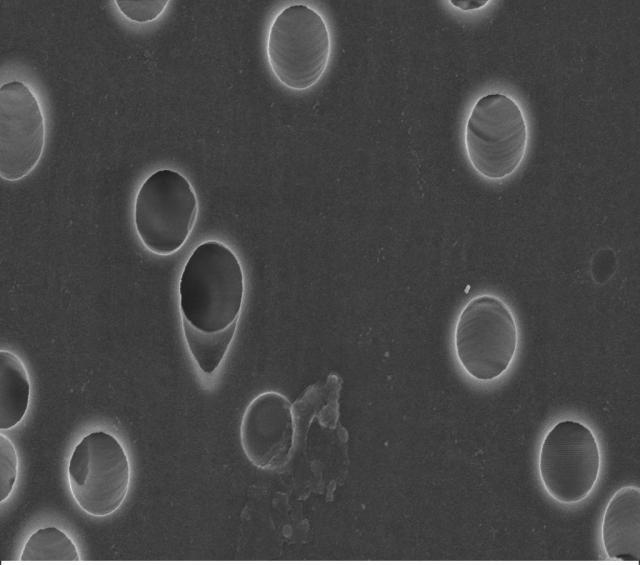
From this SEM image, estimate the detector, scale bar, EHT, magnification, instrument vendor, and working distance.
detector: InLens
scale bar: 1000 nm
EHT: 3 kV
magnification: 40 K X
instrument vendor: Zeiss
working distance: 3 mm